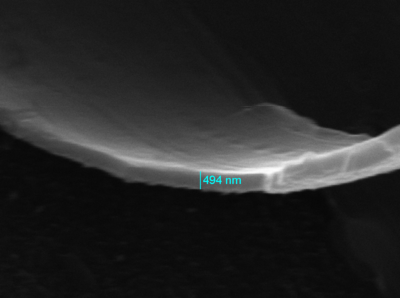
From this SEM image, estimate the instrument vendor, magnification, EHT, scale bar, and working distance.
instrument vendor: other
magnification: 11.8 K X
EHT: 25 kV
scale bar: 2000 nm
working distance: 15 mm